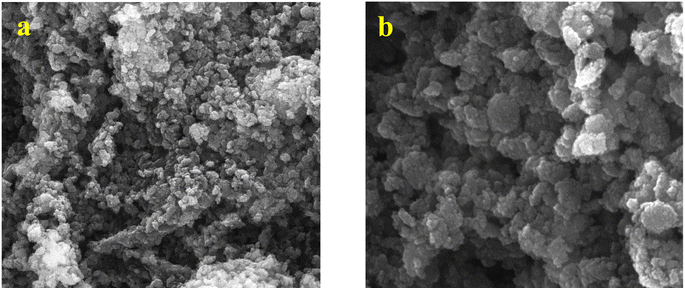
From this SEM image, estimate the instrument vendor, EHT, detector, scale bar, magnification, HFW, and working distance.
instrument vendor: Tescan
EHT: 30 kV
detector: SE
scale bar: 1000 nm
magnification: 63.5 K X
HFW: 4.36 µm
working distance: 7.39 mm